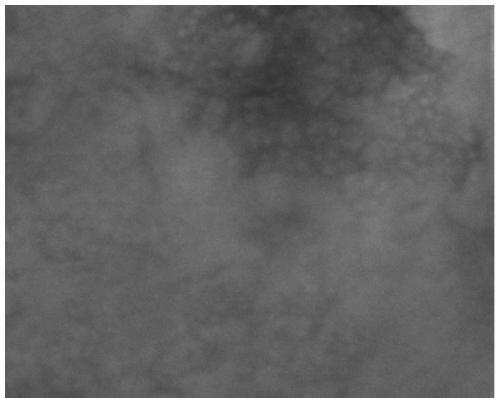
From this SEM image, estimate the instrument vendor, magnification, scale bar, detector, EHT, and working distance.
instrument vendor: FEI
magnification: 200 K X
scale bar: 500 nm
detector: ETD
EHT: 10 kV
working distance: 9.8 mm